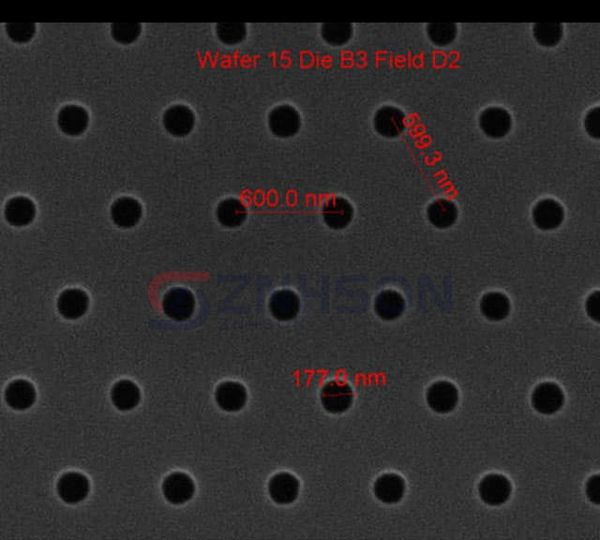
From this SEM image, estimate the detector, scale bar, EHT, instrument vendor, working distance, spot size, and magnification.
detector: ETD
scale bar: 1000 nm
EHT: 10 kV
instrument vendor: FEI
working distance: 10.3 mm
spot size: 1.5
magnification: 75 K X